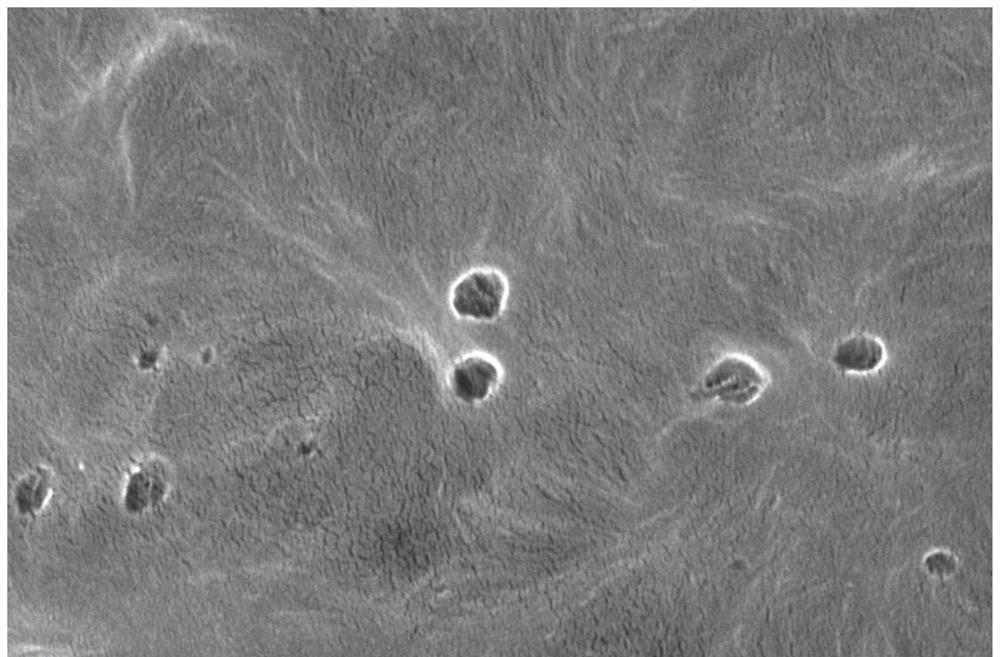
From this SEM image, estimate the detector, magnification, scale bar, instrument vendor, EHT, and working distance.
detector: T2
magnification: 100 K X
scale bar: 1000 nm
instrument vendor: Thermo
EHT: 5 kV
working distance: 17.2998 mm